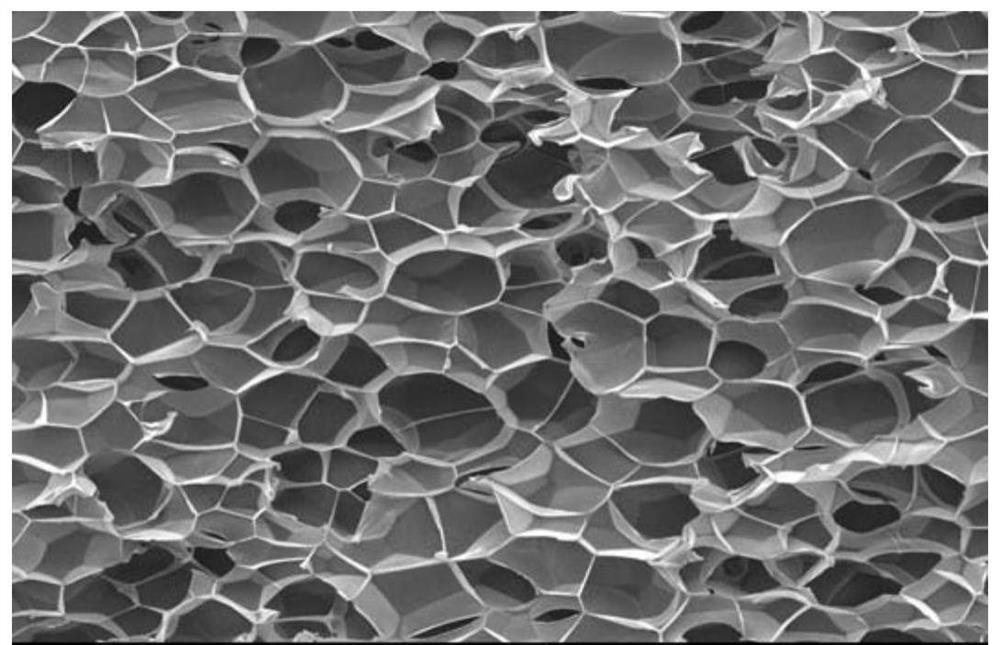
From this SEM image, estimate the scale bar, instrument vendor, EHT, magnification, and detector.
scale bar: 100000 nm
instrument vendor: JEOL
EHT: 10 kV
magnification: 0.2 K X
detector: SEI